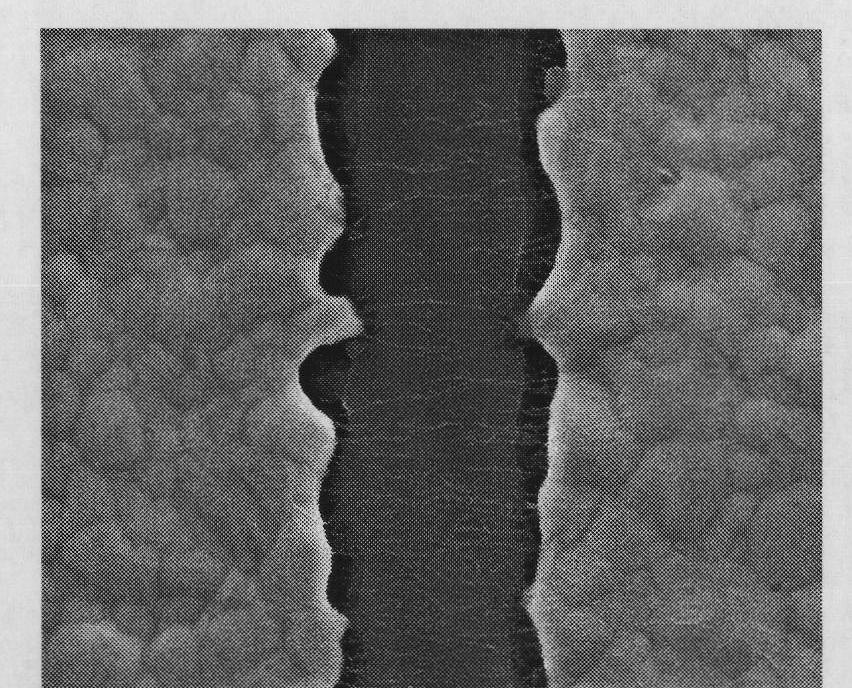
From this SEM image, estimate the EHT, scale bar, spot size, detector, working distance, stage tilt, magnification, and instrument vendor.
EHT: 10 kV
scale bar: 4000 nm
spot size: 3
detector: ETD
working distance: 8.8 mm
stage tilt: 0°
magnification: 24 K X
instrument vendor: FEI